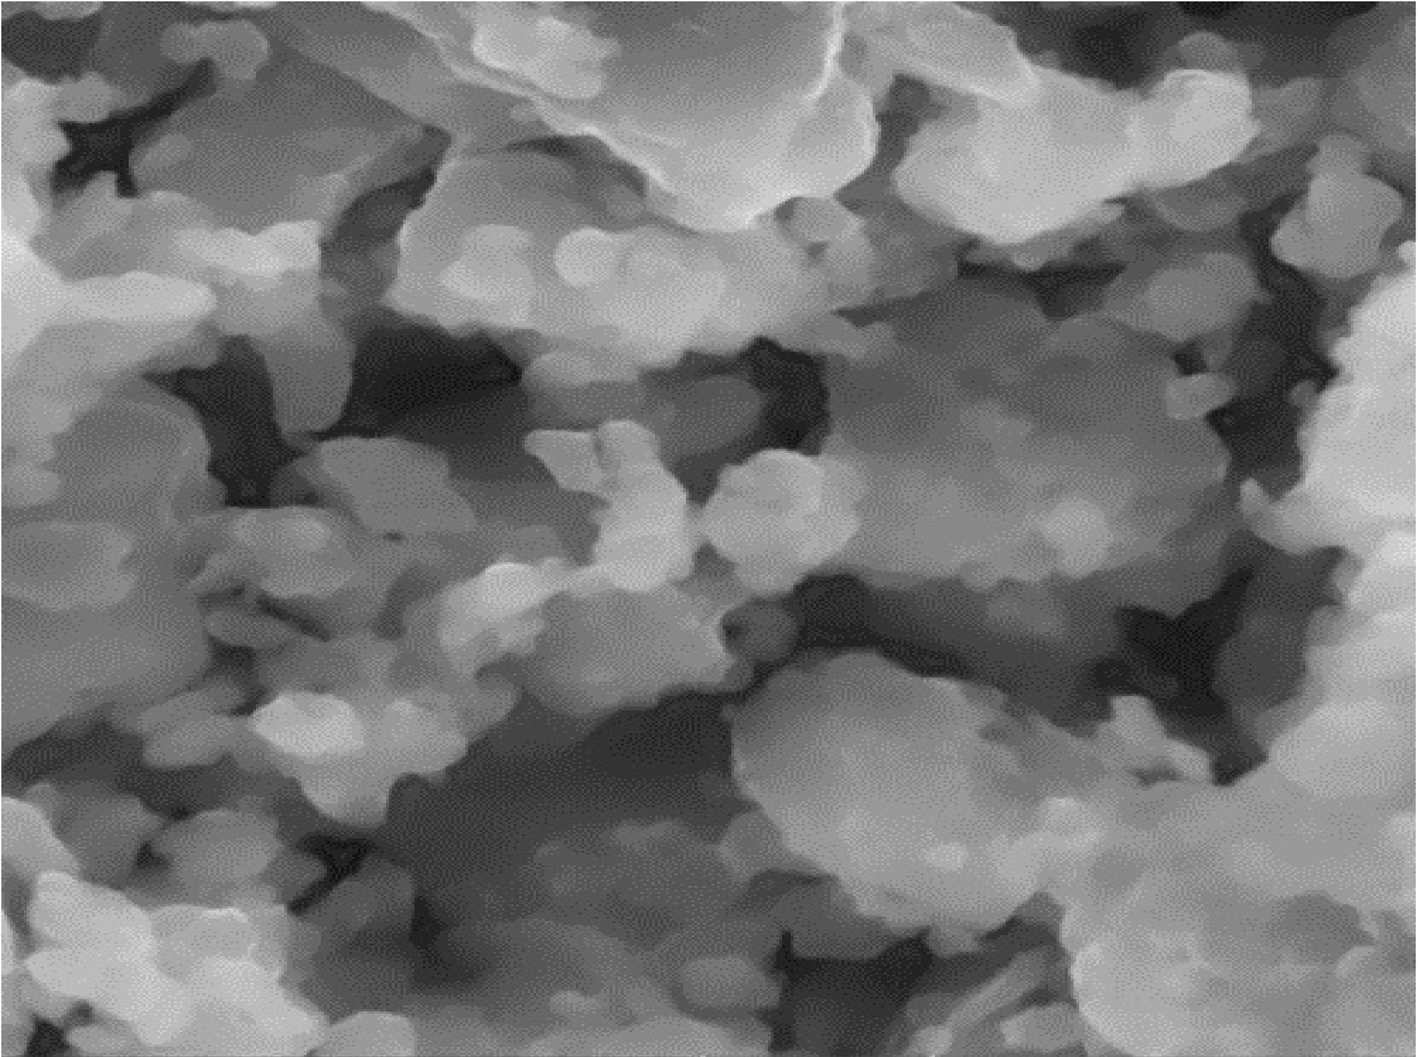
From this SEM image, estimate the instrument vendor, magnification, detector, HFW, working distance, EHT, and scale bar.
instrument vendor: Tescan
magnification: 10 K X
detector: SE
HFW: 12.7 µm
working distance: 14.58 mm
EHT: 15 kV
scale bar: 2000 nm